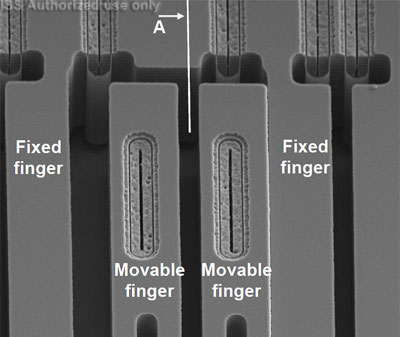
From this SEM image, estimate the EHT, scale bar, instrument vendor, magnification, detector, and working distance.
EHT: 5 kV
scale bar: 10000 nm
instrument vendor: FEI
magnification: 3.501 K X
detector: ETD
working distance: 4.4 mm